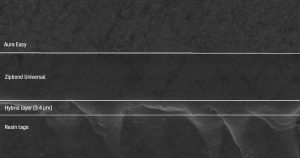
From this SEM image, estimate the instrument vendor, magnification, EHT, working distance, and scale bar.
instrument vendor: JEOL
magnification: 2 K X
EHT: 15 kV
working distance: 21 mm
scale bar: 10000 nm